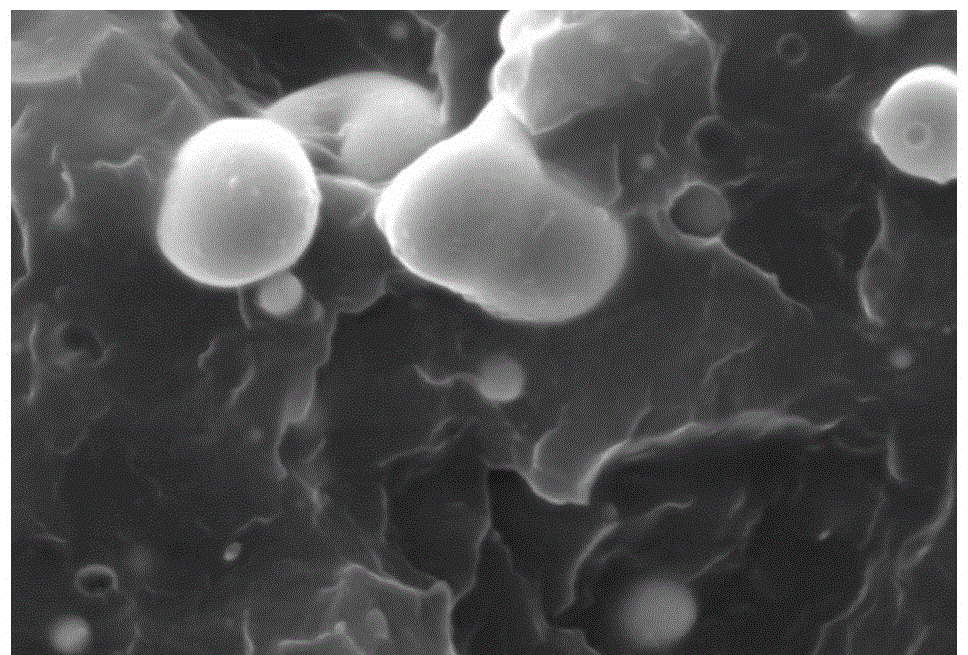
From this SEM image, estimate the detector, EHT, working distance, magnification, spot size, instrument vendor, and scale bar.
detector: SEI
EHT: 30 kV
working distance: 11 mm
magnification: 2 K X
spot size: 40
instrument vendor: JEOL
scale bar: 10000 nm